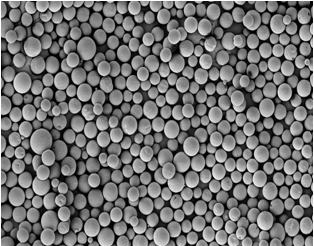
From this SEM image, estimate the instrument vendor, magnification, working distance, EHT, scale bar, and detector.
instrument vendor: Zeiss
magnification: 0.5 K X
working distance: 10.5 mm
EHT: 5 kV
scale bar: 20000 nm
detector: SE2